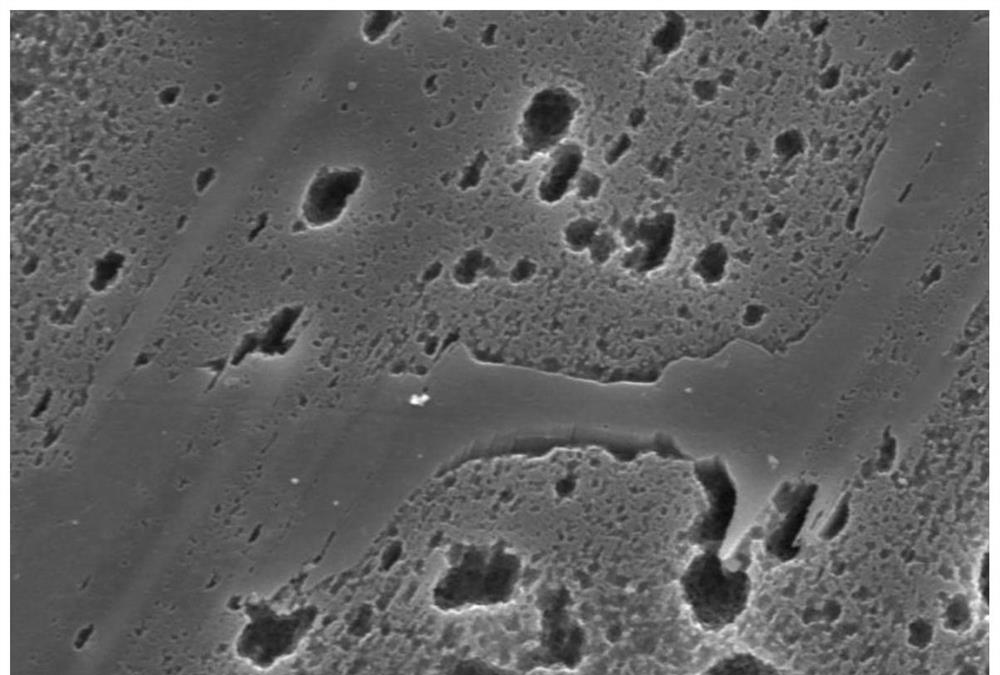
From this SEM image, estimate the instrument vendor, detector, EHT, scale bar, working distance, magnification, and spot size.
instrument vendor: other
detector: SE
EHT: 15 kV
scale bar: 10000 nm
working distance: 11.4 mm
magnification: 5 K X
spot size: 12